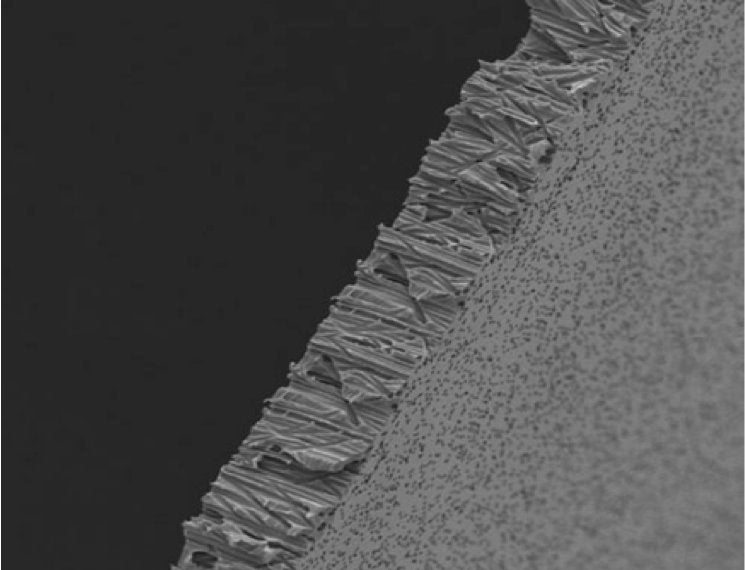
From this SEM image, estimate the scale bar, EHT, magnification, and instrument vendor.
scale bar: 10000 nm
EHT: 5 kV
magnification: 1 K X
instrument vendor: JEOL